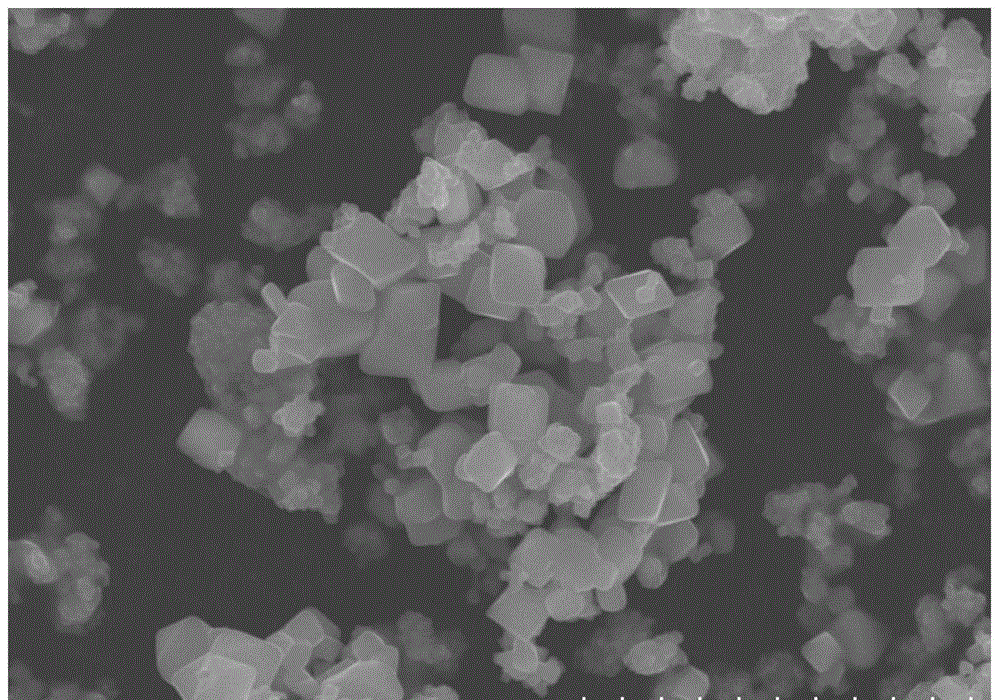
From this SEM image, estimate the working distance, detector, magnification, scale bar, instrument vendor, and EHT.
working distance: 15.6 mm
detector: SE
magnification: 5 K X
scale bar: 10000 nm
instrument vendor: Hitachi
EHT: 20 kV